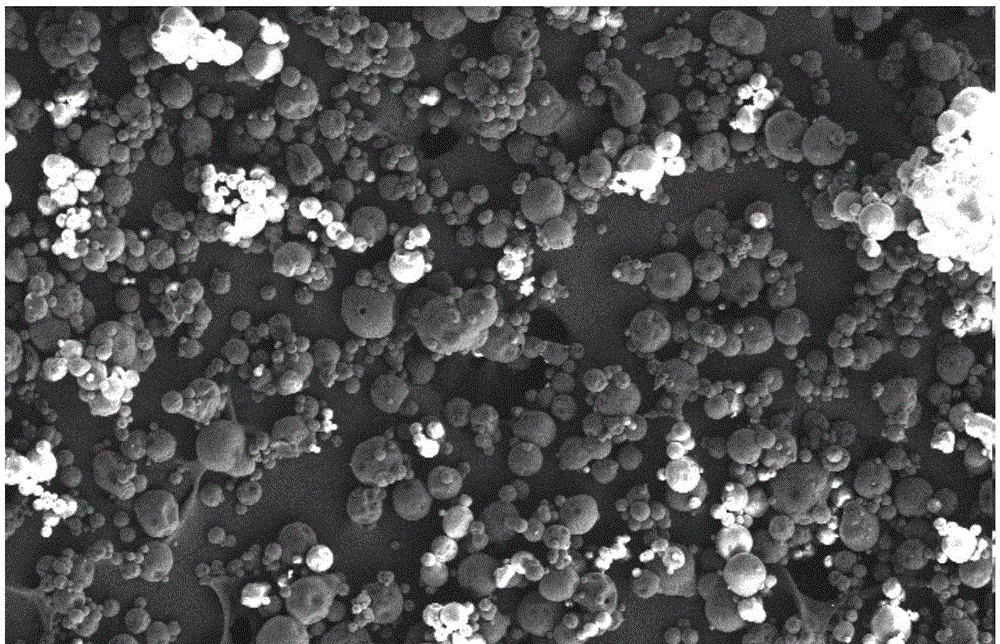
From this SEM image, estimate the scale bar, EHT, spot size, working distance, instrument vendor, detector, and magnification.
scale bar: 200000 nm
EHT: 15 kV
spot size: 3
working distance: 9.8 mm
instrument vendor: FEI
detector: SE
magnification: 0.1 K X